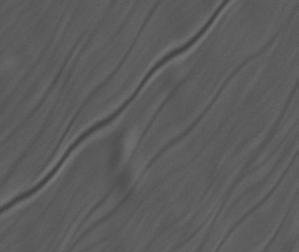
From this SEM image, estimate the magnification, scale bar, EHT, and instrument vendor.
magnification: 20 K X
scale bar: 500 nm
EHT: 10 kV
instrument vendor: JEOL